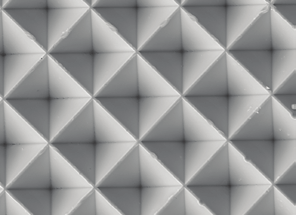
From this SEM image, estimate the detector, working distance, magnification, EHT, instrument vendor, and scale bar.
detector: SEI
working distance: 25.1 mm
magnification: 1.1 K X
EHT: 10 kV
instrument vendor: JEOL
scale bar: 10000 nm